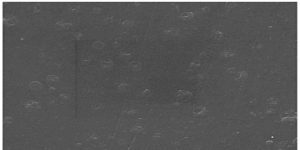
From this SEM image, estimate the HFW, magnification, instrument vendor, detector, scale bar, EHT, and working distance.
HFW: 27.7 µm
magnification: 10 K X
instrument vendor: Tescan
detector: SE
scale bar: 5000 nm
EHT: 15 kV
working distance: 18.07 mm